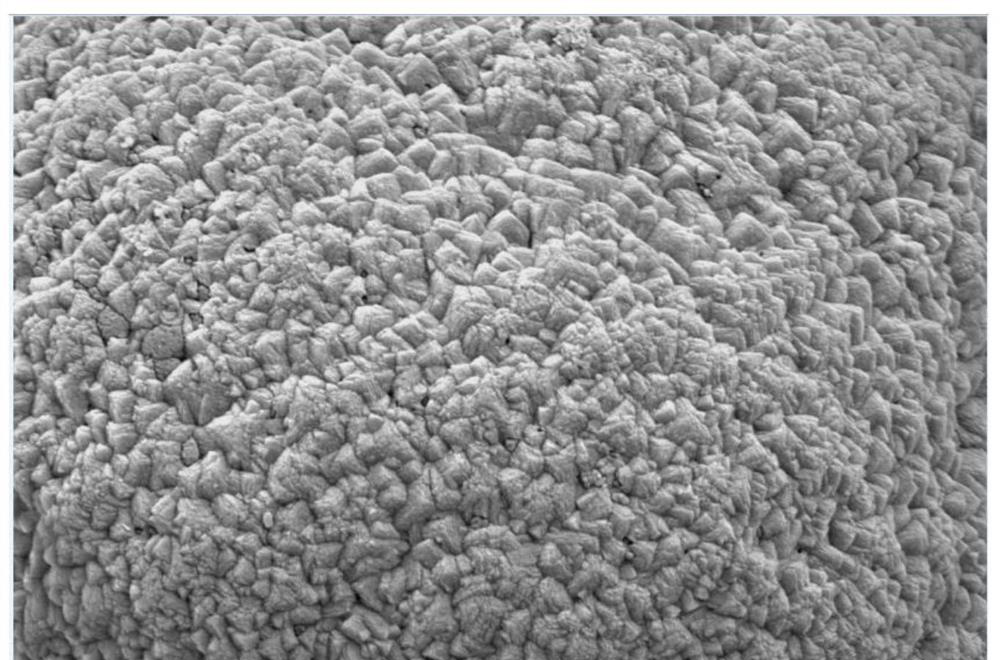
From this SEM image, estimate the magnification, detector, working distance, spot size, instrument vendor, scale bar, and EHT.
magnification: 30 K X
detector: ETD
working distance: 10.6 mm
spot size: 3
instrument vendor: FEI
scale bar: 5000 nm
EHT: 5 kV